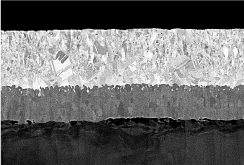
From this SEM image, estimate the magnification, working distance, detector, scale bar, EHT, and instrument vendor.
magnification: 10 K X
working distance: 8.5 mm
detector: PDBSE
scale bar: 5000 nm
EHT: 6 kV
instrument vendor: Hitachi